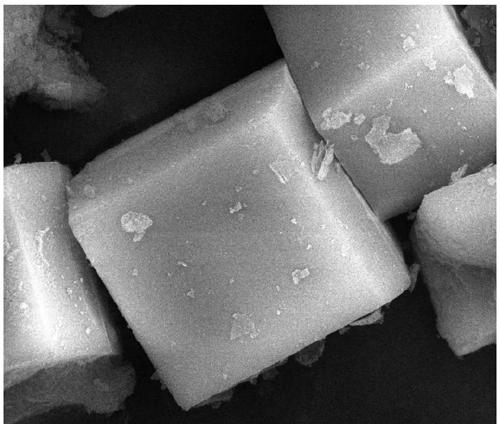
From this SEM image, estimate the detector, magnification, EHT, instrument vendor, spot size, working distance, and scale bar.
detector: ETD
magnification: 10 K X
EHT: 20 kV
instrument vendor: FEI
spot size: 3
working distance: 10 mm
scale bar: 5000 nm